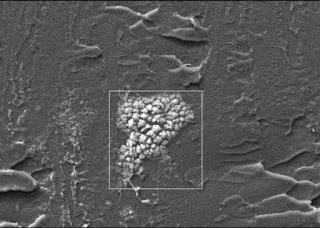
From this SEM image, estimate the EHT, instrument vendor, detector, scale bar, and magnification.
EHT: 10 kV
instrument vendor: other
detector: RBSE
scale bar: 20000 nm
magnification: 0.5 K X